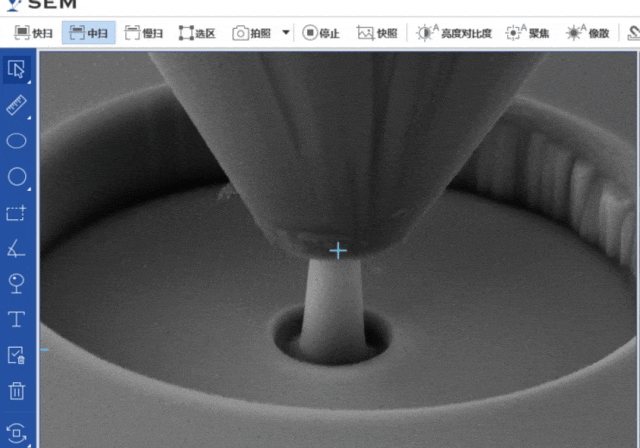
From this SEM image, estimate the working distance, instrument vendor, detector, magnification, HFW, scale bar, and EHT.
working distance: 15.02 mm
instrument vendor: other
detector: ETD-SE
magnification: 4.5 K X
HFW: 28.47 µm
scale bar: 2000 nm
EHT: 15 kV